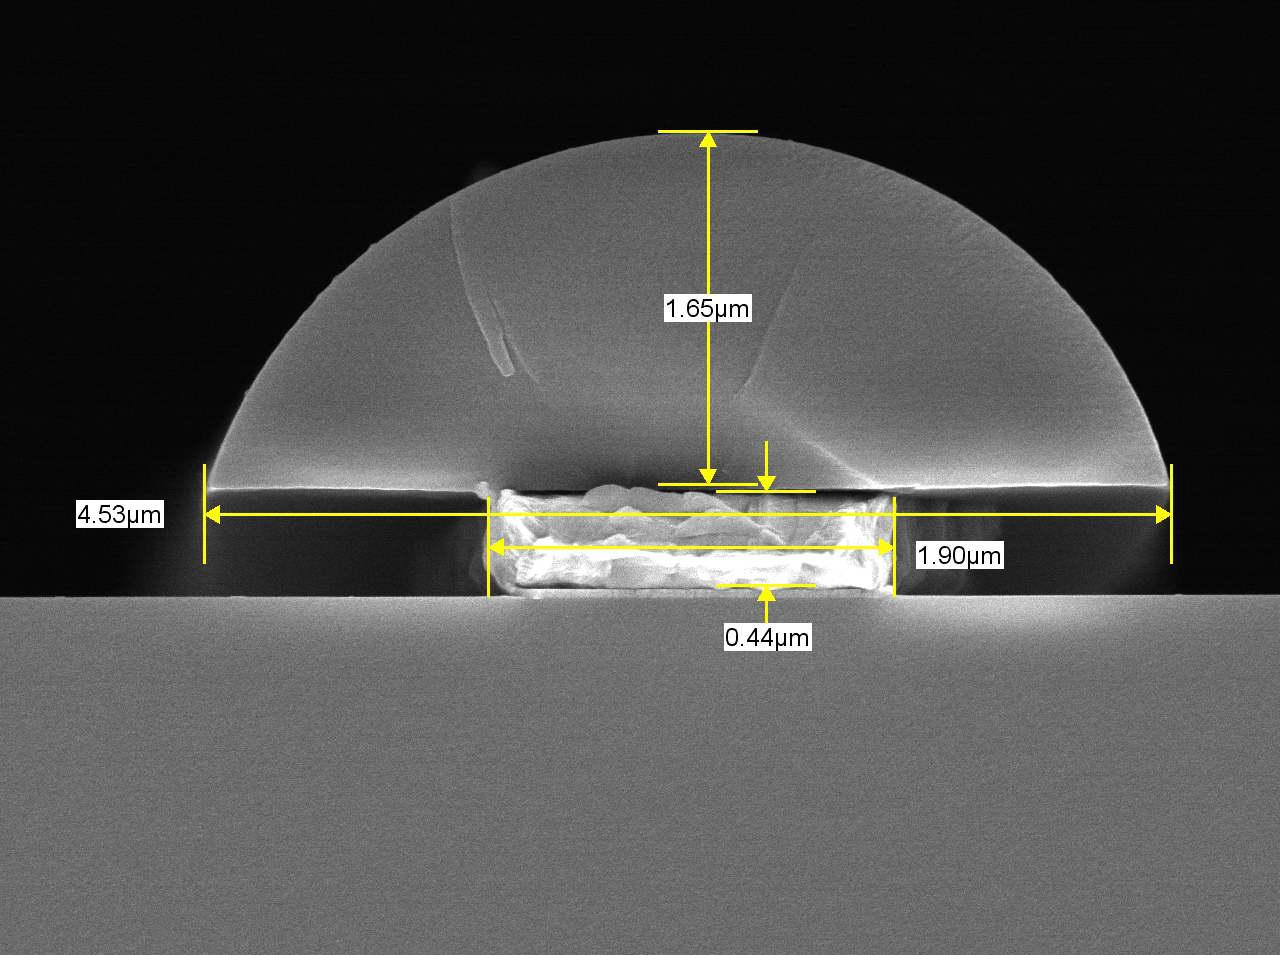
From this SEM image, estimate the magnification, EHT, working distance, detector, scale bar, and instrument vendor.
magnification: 20 K X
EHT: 5 kV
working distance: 6.1 mm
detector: SEM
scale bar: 1000 nm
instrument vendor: JEOL